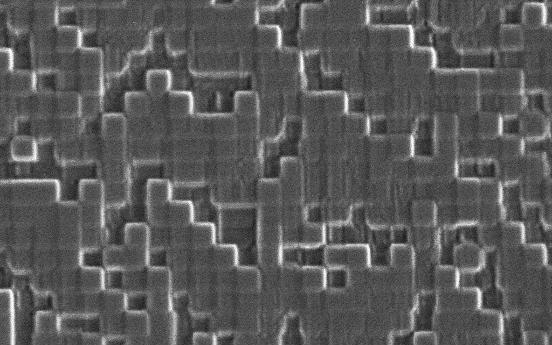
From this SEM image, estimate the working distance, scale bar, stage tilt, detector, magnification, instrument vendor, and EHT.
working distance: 11.2 mm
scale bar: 1000 nm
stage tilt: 0°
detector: SE2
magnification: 10 K X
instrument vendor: Zeiss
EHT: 3 kV